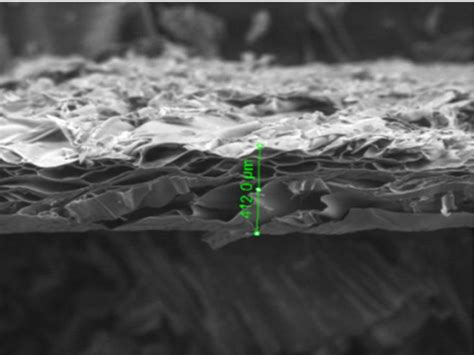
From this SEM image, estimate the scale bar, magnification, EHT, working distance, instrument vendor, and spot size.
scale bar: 1000000 nm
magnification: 0.15 K X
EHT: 20 kV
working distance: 11.4 mm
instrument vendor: FEI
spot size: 4